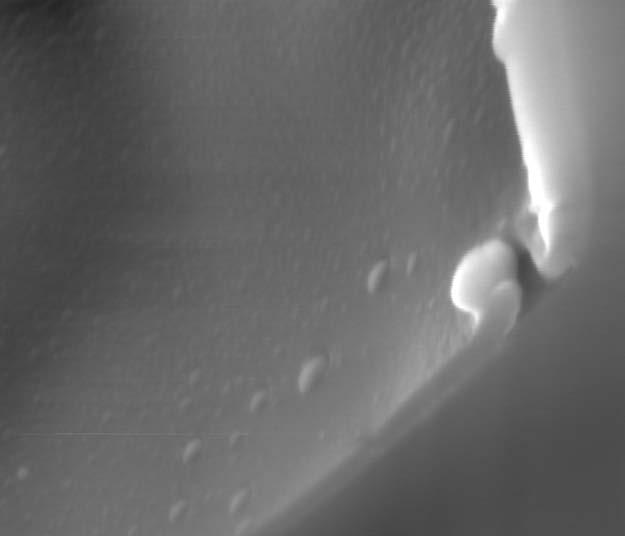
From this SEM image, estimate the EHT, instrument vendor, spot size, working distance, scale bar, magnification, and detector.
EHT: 15 kV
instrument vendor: FEI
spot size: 1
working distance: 10 mm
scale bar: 1000 nm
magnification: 59.996 K X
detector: ETD